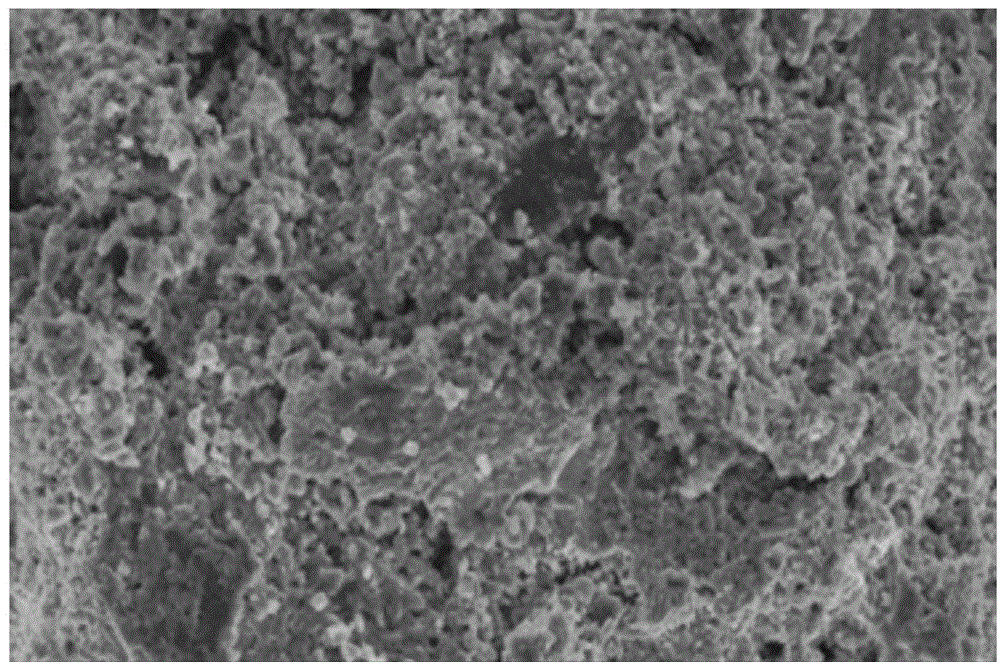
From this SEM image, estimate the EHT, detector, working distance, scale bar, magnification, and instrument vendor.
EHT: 5 kV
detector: SEI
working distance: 9 mm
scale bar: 1000 nm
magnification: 5 K X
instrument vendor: JEOL